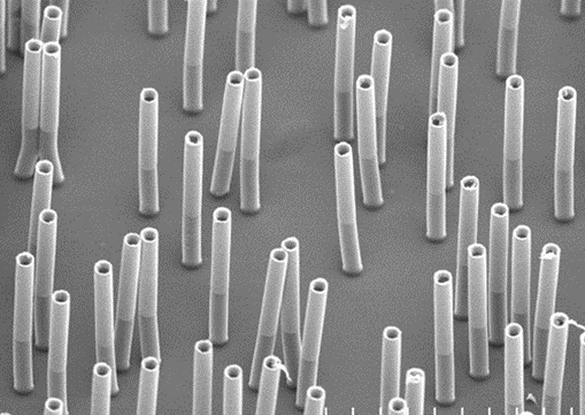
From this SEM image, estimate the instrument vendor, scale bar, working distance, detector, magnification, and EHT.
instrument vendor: Hitachi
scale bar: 30000 nm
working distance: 10.7 mm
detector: SE(M)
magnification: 1.8 K X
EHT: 7 kV